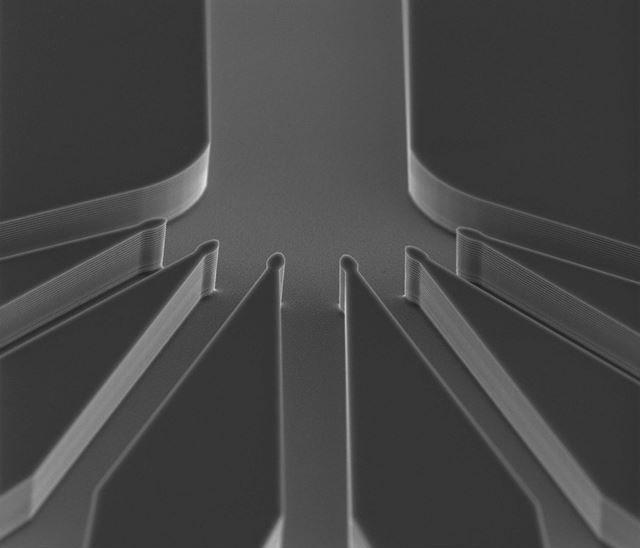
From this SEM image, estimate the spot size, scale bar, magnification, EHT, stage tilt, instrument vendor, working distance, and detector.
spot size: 4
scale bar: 50000 nm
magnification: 0.784 K X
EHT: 20 kV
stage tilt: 29.5°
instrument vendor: FEI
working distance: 12.18 mm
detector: Etd